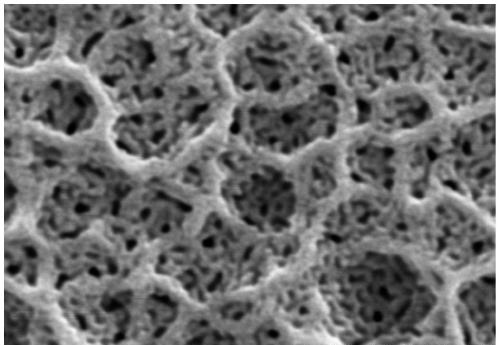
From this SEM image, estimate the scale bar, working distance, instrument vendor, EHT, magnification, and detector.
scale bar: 500 nm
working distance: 17 mm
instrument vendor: Hitachi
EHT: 15 kV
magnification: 100 K X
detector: SE(M)